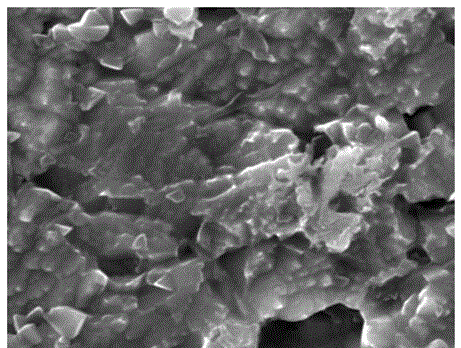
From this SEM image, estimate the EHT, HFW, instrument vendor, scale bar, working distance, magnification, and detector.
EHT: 30 kV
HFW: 10.4 µm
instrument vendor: Tescan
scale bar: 2000 nm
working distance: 15.48 mm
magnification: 20 K X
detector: SE